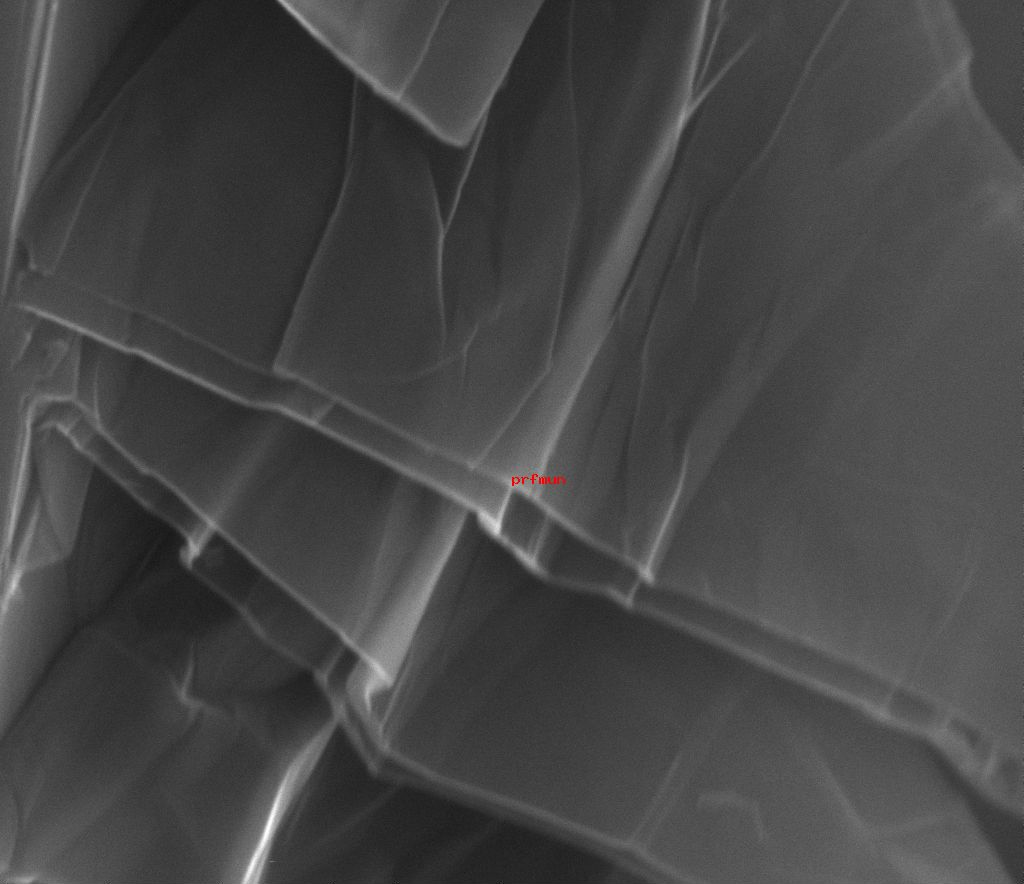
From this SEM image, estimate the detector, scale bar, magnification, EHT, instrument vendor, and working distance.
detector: ETD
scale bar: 5000 nm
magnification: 28 K X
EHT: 28 kV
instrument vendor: FEI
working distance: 10 mm